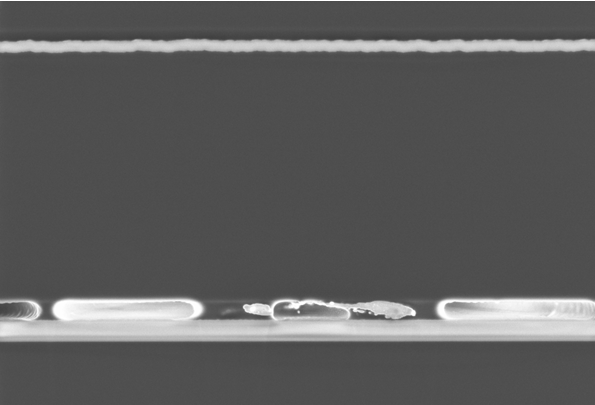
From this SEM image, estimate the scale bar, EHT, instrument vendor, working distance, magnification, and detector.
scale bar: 1000 nm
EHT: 3 kV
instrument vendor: Zeiss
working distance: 4.4 mm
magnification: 6.64 K X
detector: InLens